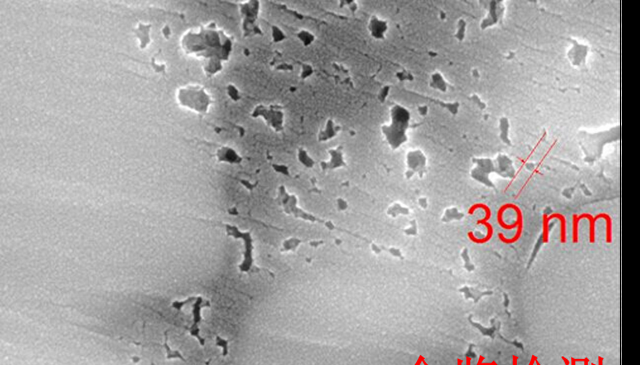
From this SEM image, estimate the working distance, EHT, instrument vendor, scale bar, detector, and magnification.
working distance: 6.03 mm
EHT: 20 kV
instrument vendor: Tescan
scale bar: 500 nm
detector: InBeam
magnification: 100 K X